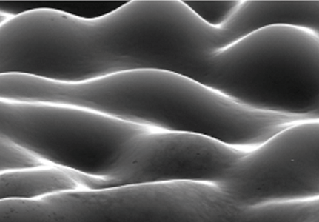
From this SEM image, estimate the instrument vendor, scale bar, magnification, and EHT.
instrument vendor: JEOL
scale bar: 20000 nm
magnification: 2.5 K X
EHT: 25 kV